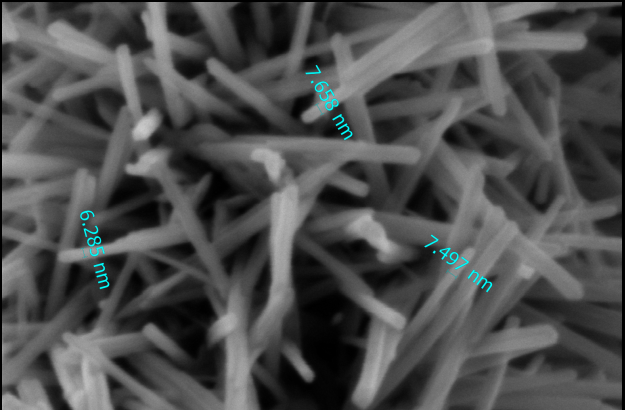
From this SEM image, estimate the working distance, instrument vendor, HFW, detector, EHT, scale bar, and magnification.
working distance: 3.3 mm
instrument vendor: Thermo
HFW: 0.423 µm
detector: T2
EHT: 2 kV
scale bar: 200 nm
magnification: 300 K X